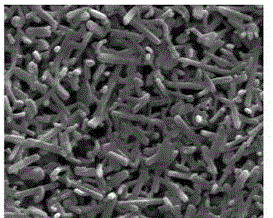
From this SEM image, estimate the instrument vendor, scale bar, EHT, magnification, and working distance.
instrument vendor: FEI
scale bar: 500 nm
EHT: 20 kV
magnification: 130 K X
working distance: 4.3 mm